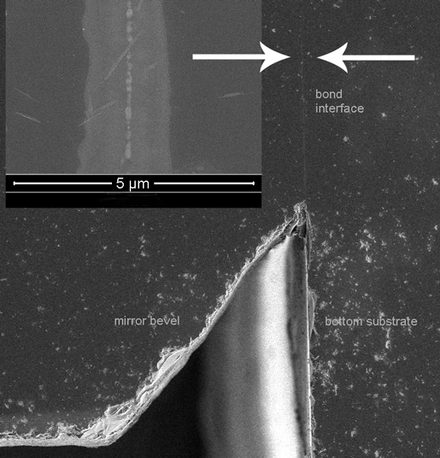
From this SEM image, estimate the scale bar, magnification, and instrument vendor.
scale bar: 300000 nm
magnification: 0.15 K X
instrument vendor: FEI